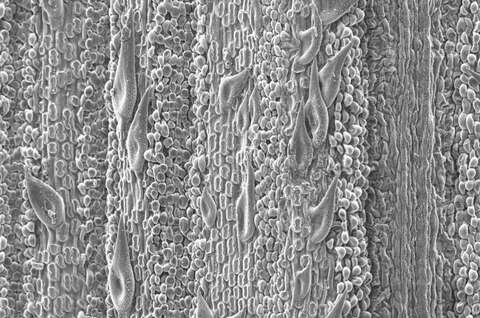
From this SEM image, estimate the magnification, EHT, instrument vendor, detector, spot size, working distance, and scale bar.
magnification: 0.2 K X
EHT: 10 kV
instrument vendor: FEI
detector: SE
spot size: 2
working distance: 9.8 mm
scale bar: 100000 nm